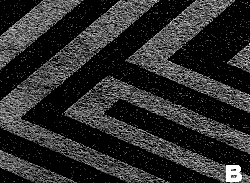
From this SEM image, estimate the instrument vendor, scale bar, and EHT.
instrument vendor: JEOL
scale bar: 100000 nm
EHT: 20 kV